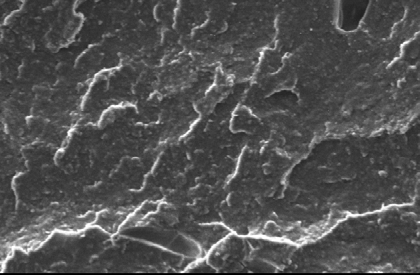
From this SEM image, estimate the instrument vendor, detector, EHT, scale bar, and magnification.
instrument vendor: JEOL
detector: SEI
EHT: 10 kV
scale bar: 10000 nm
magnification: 1 K X